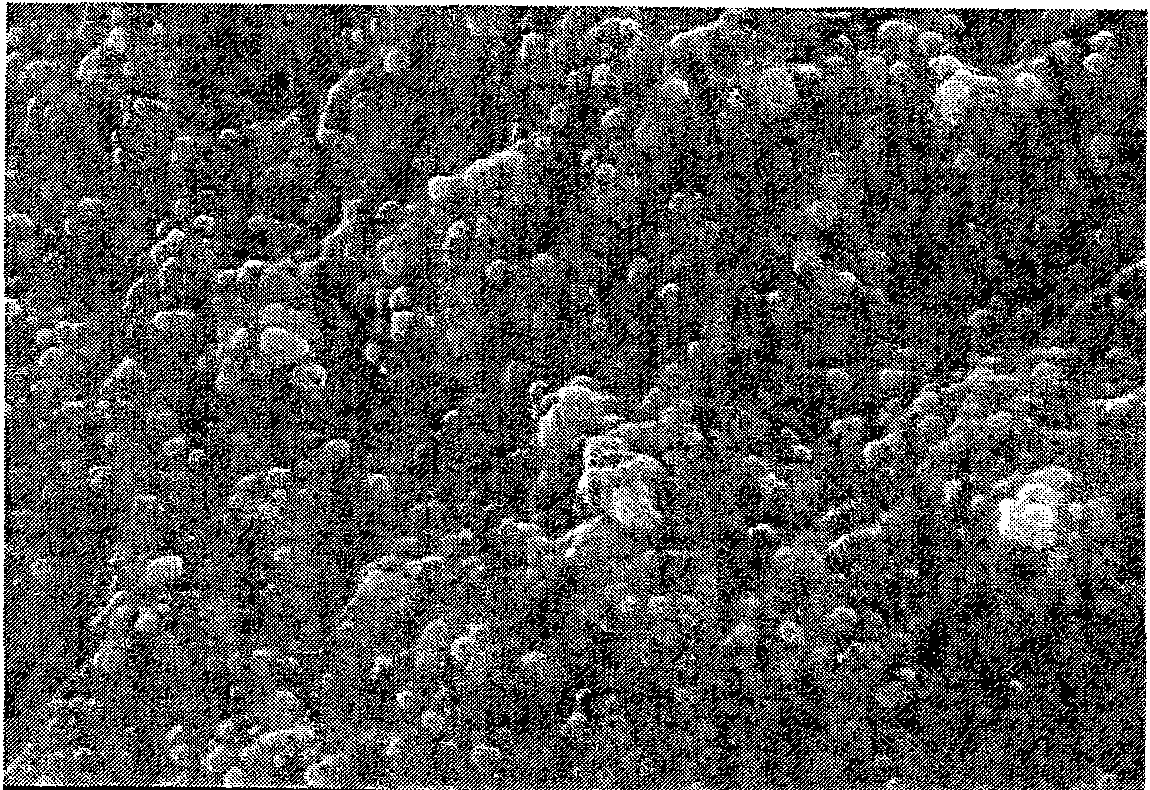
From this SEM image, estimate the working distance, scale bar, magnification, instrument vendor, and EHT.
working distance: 11.9 mm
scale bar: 2000 nm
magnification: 20 K X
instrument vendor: Hitachi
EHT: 20 kV